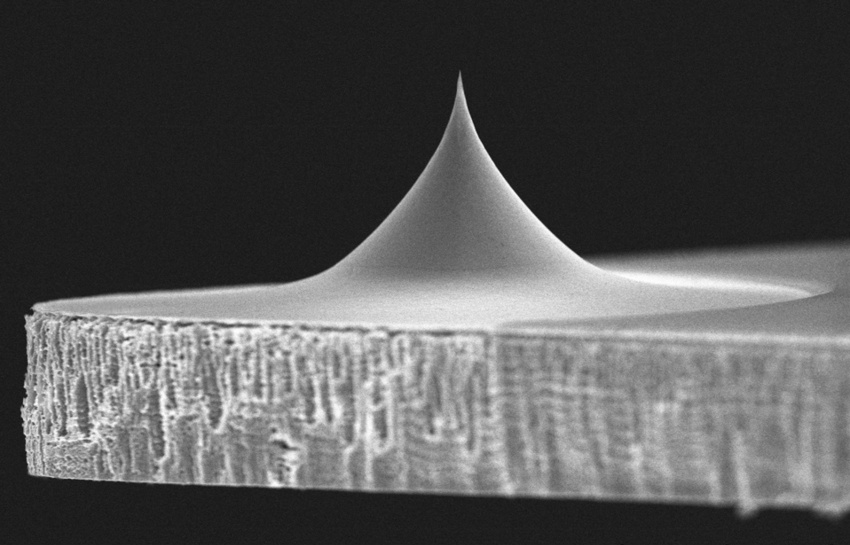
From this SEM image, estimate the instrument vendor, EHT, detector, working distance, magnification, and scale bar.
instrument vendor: Hitachi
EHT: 4 kV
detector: SE(UL)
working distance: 7.2 mm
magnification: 6 K X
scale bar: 5000 nm